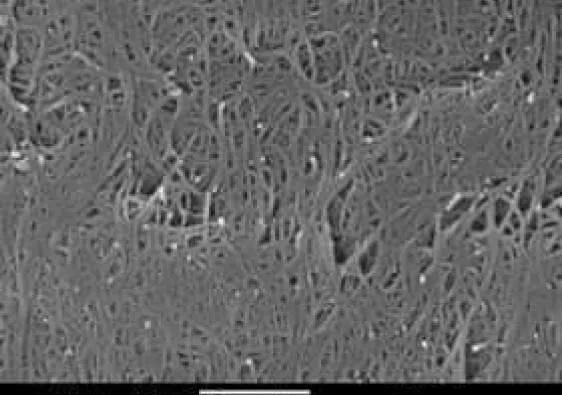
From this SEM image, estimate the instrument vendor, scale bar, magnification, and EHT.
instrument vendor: JEOL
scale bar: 1000 nm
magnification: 25 K X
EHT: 20 kV